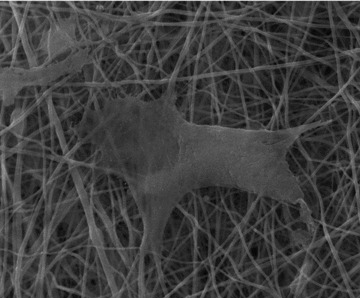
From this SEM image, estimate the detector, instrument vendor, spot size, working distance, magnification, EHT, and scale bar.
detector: SE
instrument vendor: FEI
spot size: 2.5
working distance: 10.1 mm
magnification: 5 K X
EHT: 20 kV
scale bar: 20000 nm